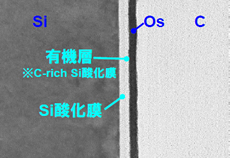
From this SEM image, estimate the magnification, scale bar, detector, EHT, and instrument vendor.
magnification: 1000 K X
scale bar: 30 nm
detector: TE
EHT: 200 kV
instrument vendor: Hitachi